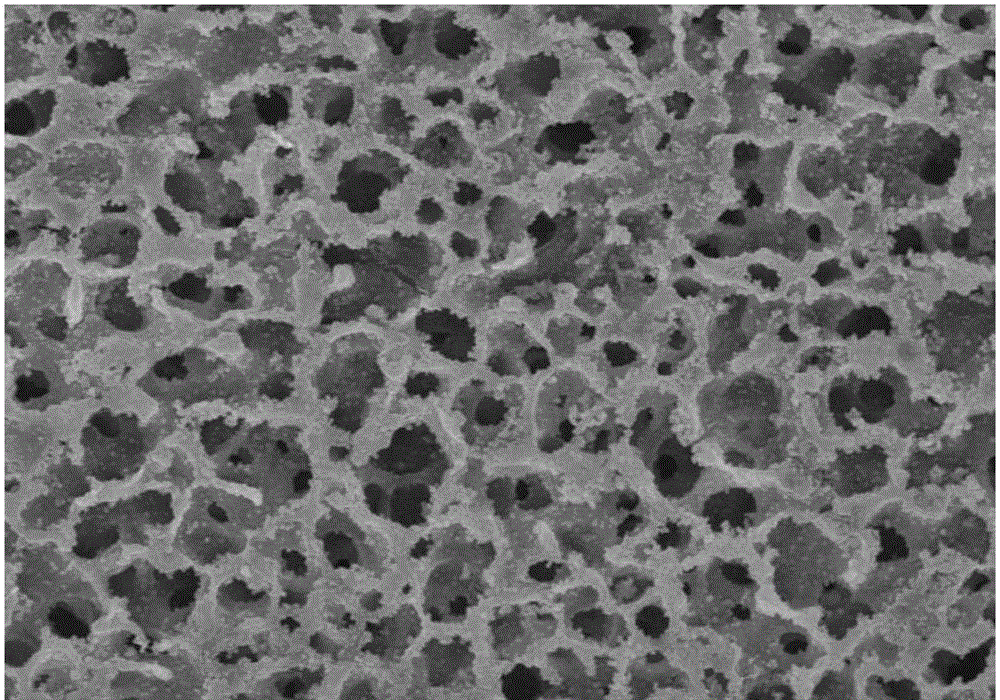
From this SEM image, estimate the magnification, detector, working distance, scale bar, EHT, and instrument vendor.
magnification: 2 K X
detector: SE(M)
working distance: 6.4 mm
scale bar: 20000 nm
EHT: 15 kV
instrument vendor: Hitachi